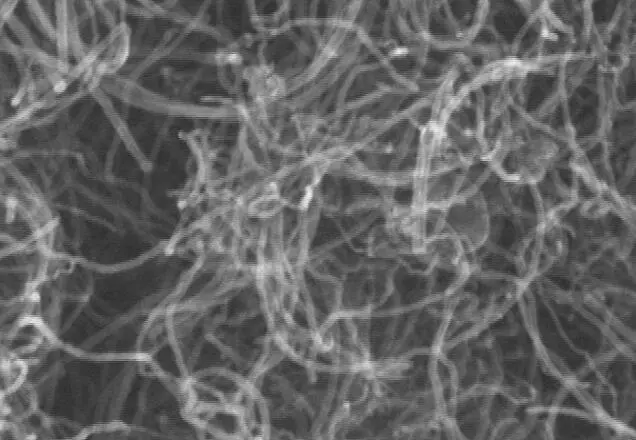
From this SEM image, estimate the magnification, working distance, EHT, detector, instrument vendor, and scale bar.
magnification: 100 K X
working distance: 9.5 mm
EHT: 15 kV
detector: SE(M)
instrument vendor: Hitachi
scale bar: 500 nm